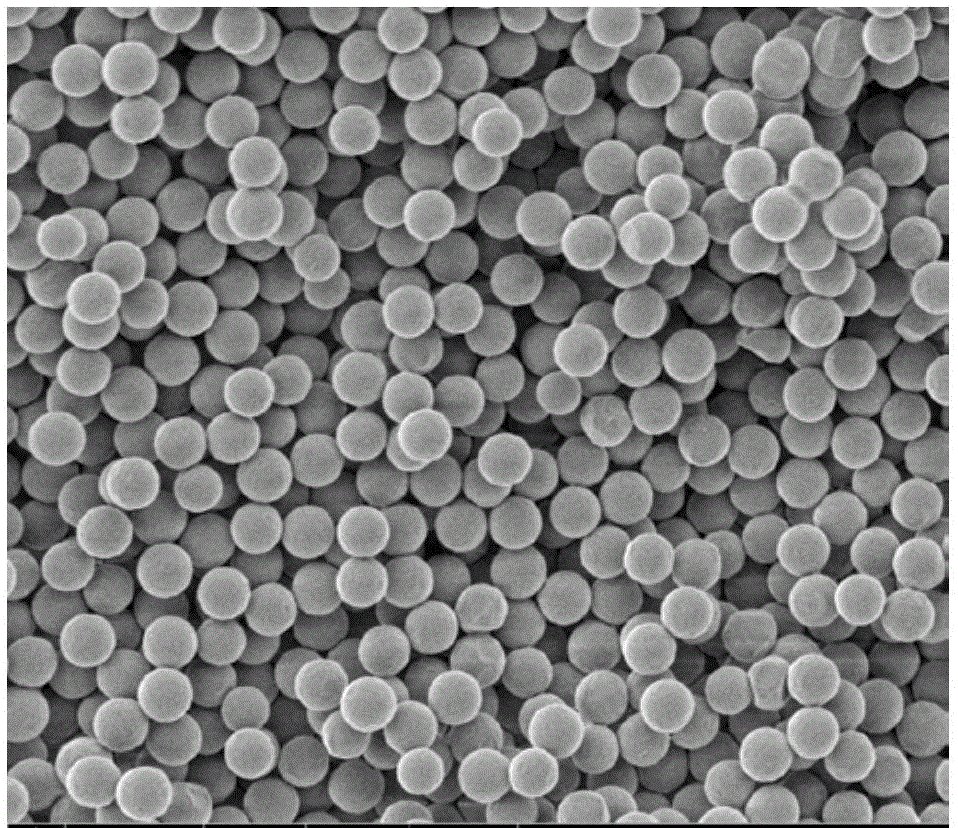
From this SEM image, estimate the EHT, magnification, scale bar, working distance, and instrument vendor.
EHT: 1 kV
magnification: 10 K X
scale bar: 5000 nm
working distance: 4.4 mm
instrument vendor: FEI